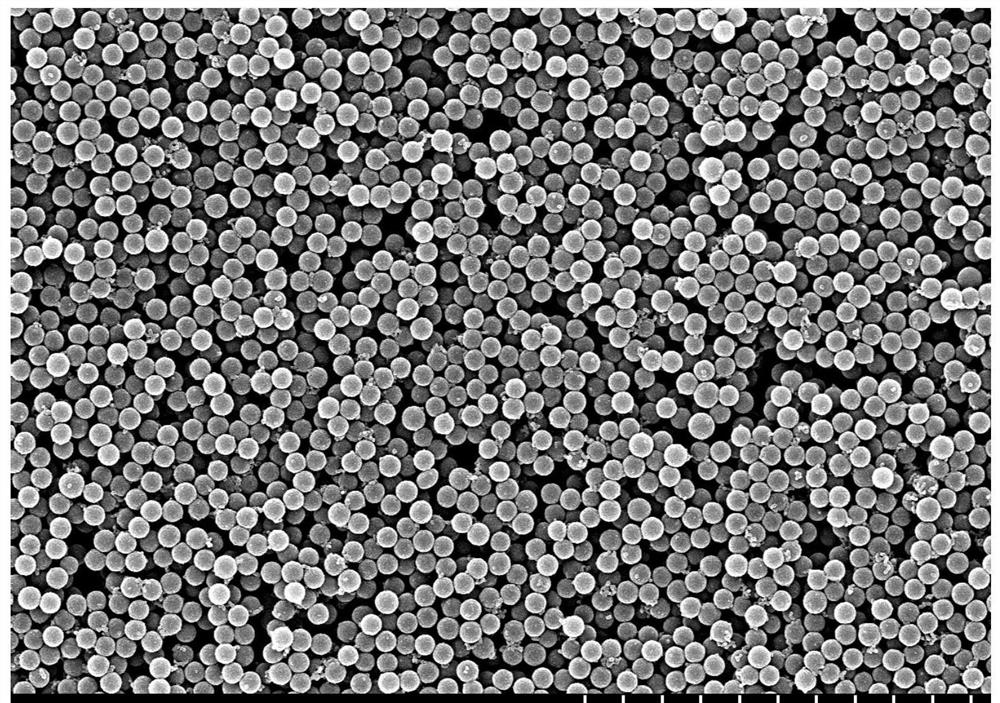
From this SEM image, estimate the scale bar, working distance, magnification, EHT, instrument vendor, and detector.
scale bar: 5000 nm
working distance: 7.4 mm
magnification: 10 K X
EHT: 10 kV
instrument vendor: Hitachi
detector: SE(M)